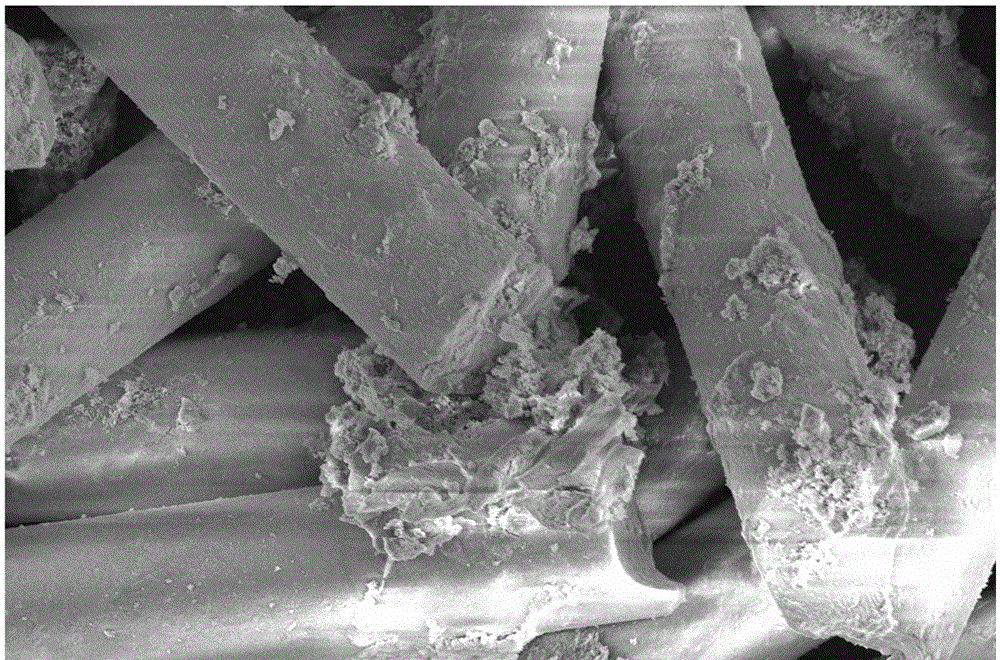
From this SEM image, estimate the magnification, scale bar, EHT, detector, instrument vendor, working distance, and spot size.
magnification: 5 K X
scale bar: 30000 nm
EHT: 5 kV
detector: ETD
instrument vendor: FEI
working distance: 5.3 mm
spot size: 3